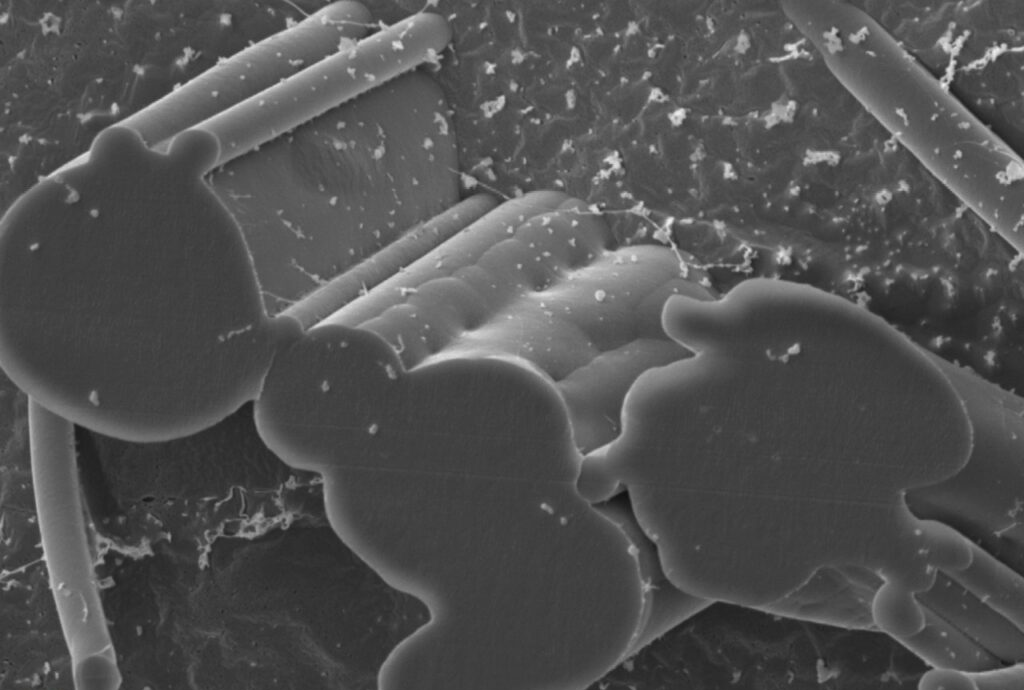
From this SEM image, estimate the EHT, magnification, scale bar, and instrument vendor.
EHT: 5 kV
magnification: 5 K X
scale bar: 5000 nm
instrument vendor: JEOL